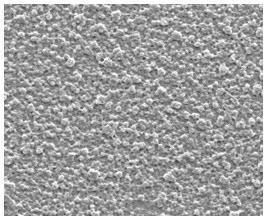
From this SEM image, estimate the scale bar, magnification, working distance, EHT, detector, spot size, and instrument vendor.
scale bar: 40000 nm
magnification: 2 K X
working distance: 5.7 mm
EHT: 10 kV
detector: ETD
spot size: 3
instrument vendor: FEI